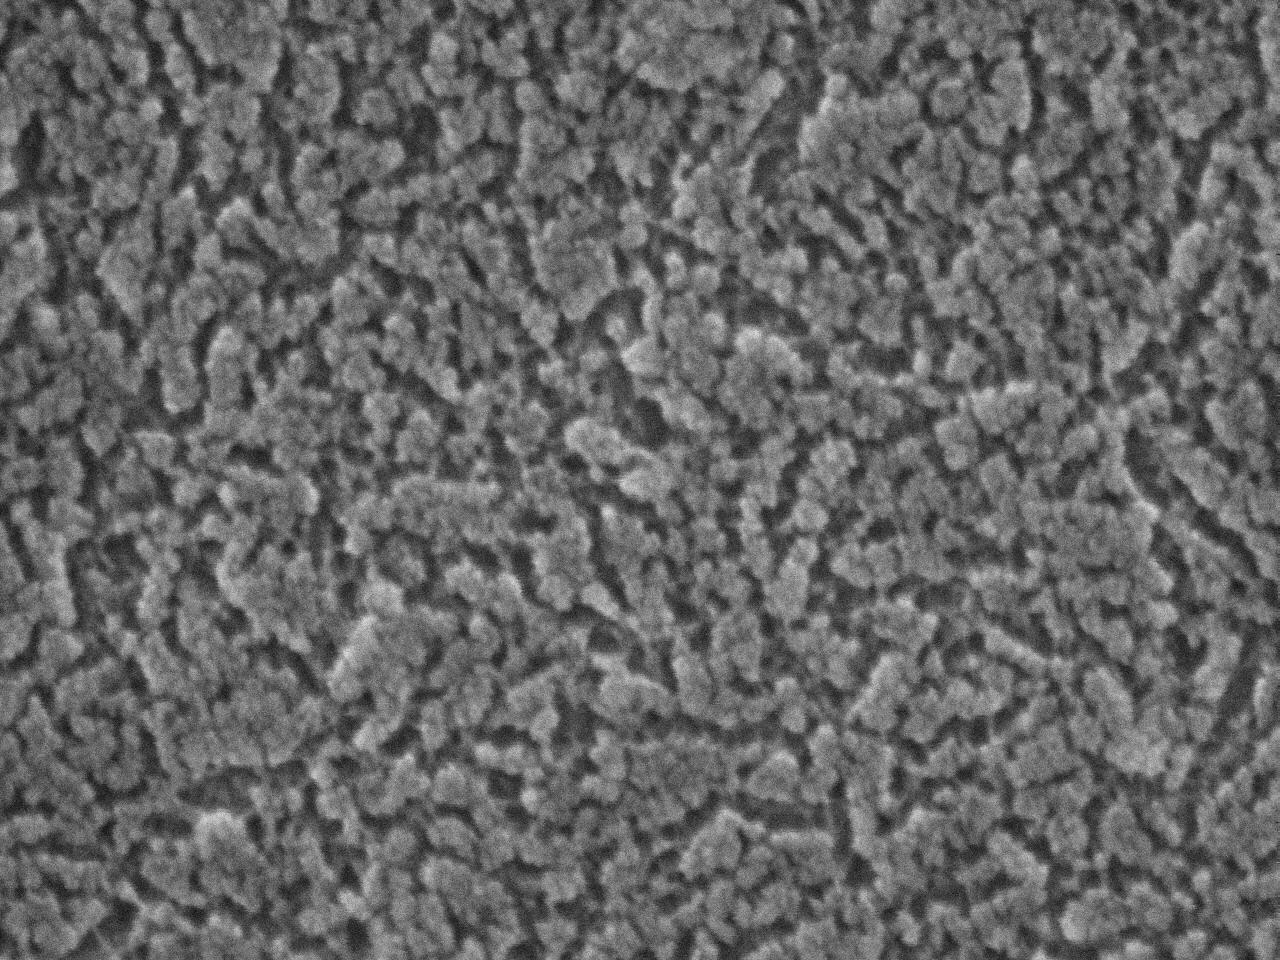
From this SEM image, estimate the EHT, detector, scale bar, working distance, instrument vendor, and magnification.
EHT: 10 kV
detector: SEI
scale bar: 100 nm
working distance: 7.8 mm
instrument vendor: JEOL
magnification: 200 K X